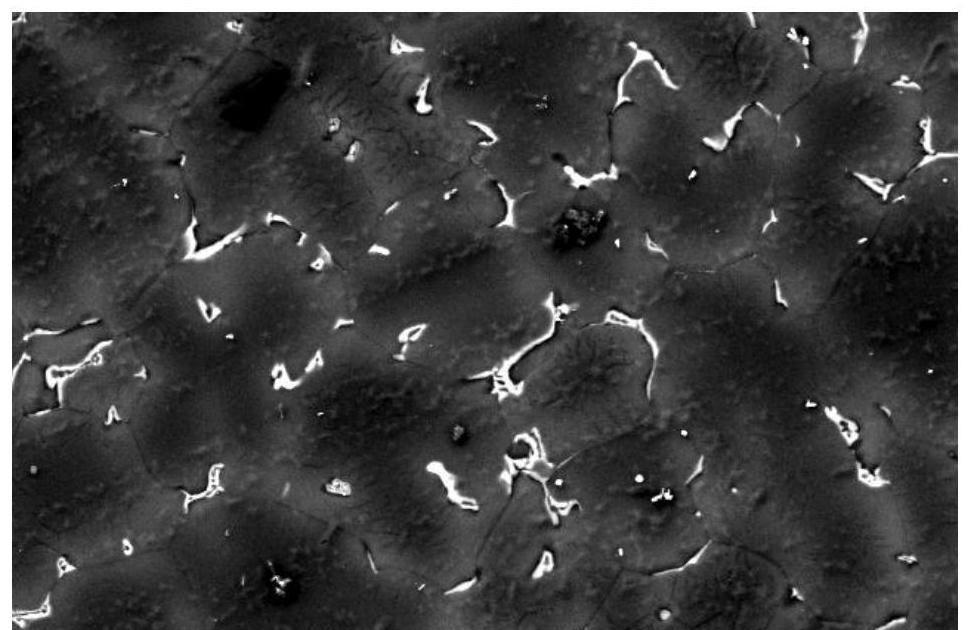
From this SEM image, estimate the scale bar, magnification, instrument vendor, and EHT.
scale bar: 50000 nm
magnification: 0.5 K X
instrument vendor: JEOL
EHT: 20 kV